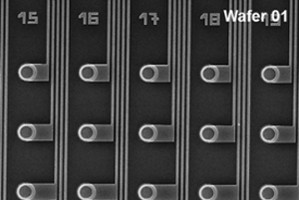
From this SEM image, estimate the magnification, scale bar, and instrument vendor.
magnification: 0.9 K X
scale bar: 33300 nm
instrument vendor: JEOL